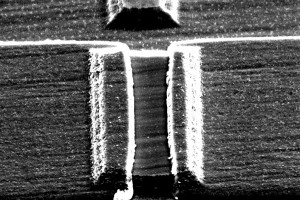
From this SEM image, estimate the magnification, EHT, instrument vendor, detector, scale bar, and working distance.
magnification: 15.27 K X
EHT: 5 kV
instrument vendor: Zeiss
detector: SE2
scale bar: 1000 nm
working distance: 16.4 mm